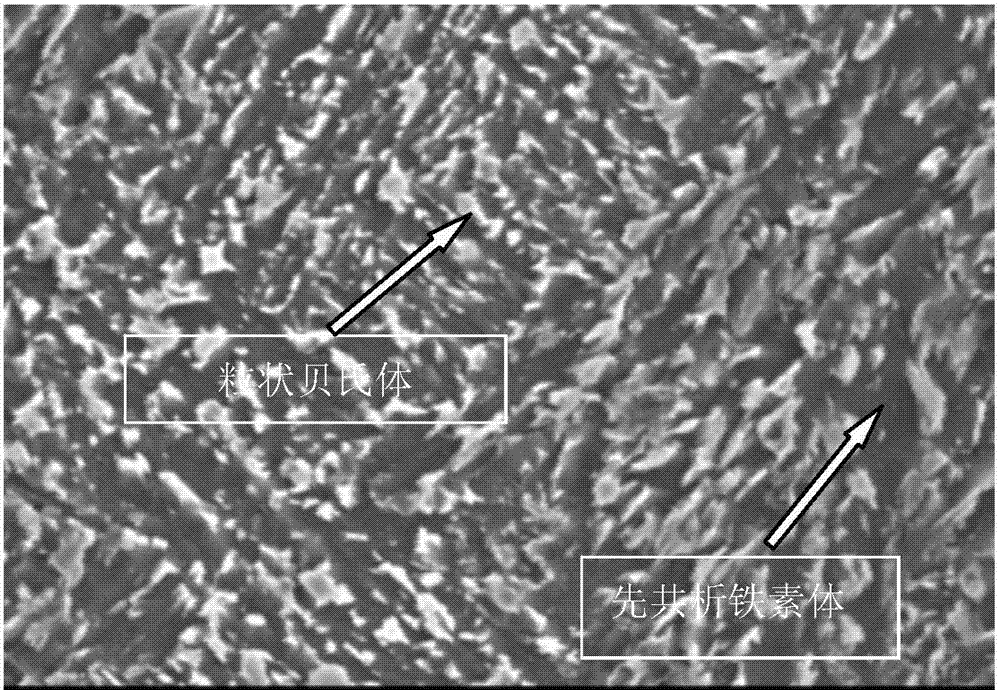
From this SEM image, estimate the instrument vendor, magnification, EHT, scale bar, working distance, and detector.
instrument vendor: JEOL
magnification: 3 K X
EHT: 25 kV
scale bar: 5000 nm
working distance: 14 mm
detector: SEI